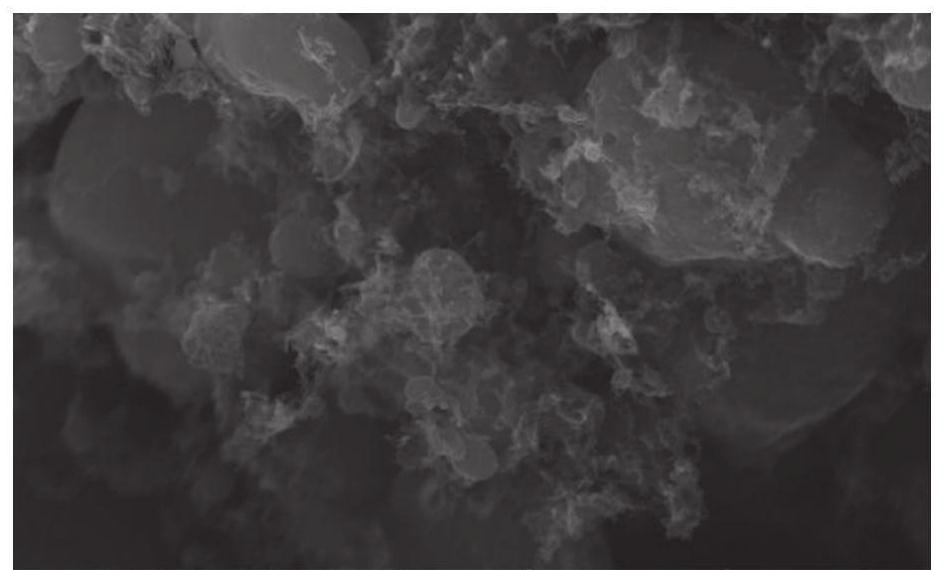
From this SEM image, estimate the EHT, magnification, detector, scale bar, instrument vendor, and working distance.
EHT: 20 kV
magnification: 20 K X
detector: In-Beam SE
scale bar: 2000 nm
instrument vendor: Tescan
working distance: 4.93 mm